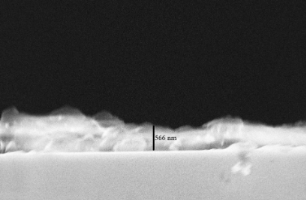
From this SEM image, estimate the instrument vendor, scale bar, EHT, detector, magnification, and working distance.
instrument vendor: Zeiss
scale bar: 1000 nm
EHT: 5 kV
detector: InLens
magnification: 11.98 K X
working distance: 2.9 mm